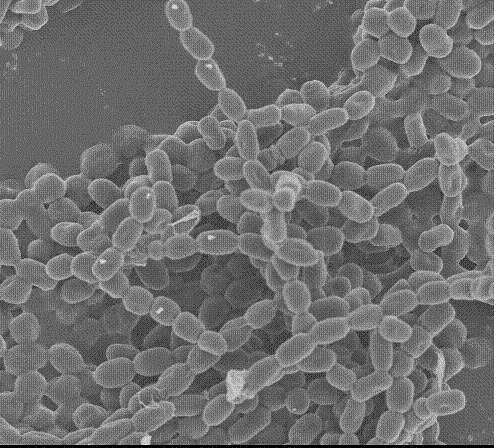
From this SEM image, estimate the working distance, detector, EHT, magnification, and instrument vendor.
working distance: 7.9 mm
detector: SE(M)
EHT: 3 kV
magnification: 8 K X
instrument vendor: Hitachi